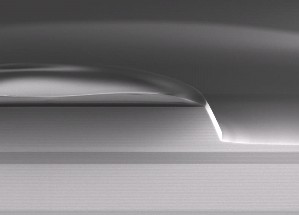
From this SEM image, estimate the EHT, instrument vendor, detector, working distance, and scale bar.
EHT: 5 kV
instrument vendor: Zeiss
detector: InLens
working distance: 2 mm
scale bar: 2000 nm